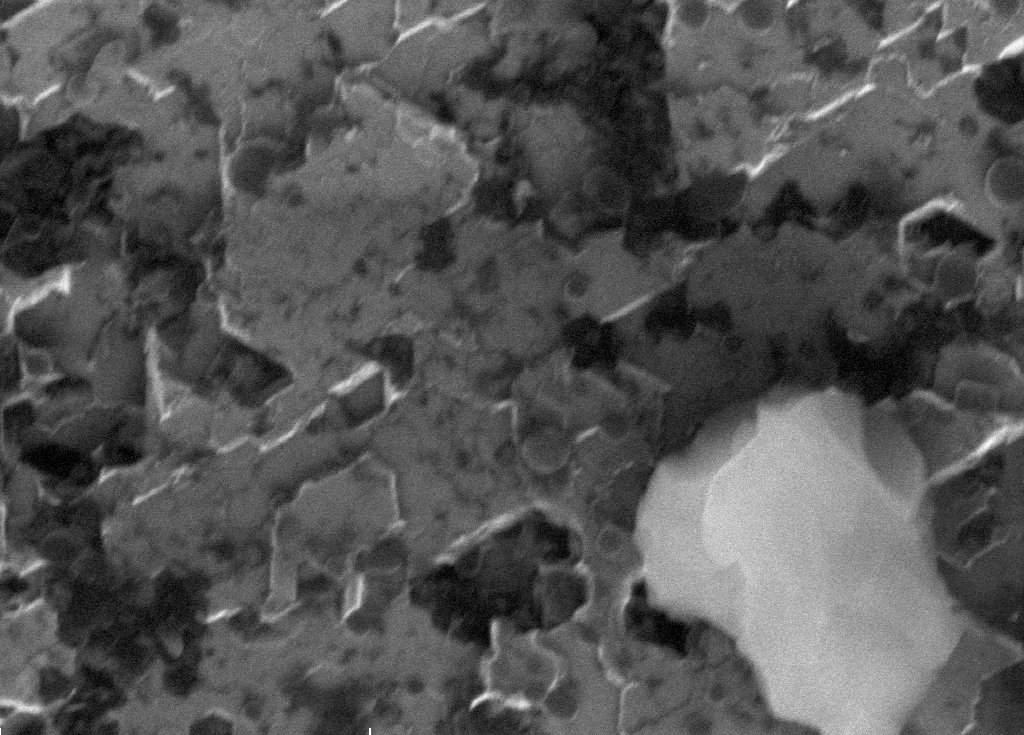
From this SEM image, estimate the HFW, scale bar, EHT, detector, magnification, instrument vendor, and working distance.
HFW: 5.54 µm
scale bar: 2000 nm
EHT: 15 kV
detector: SE, BSE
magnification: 50 K X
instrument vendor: Tescan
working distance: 15 mm